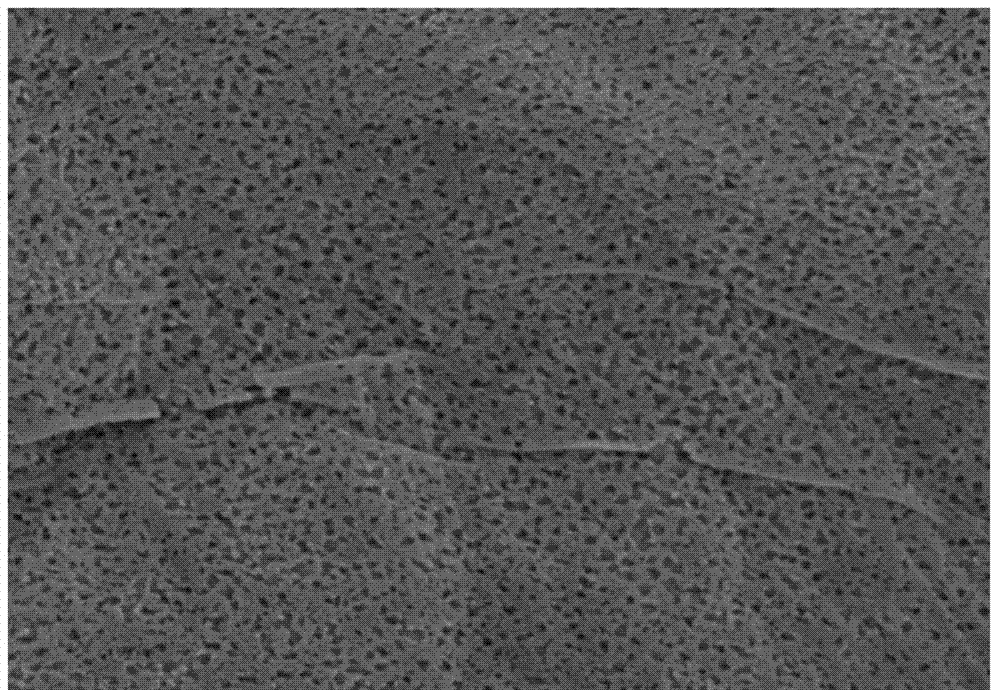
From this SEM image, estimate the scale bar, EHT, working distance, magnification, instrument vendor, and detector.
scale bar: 2000 nm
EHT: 5 kV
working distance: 7.7 mm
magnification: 25 K X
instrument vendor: Hitachi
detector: SE(M)